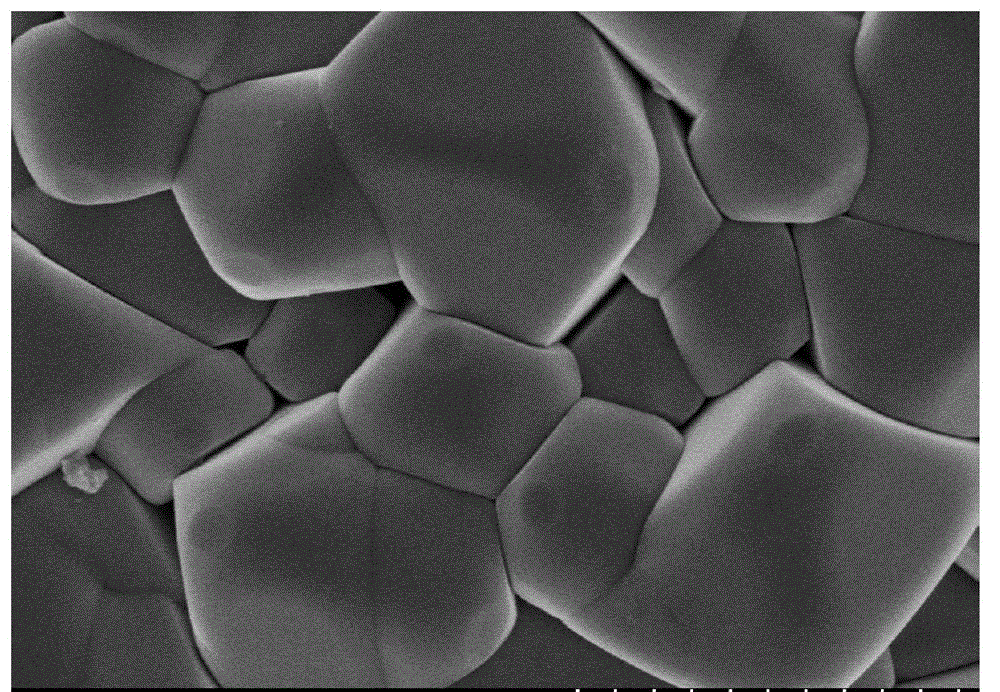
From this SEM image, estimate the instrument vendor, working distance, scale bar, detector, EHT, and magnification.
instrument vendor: Hitachi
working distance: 8 mm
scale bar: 1000 nm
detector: SE(M)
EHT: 3 kV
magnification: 50 K X